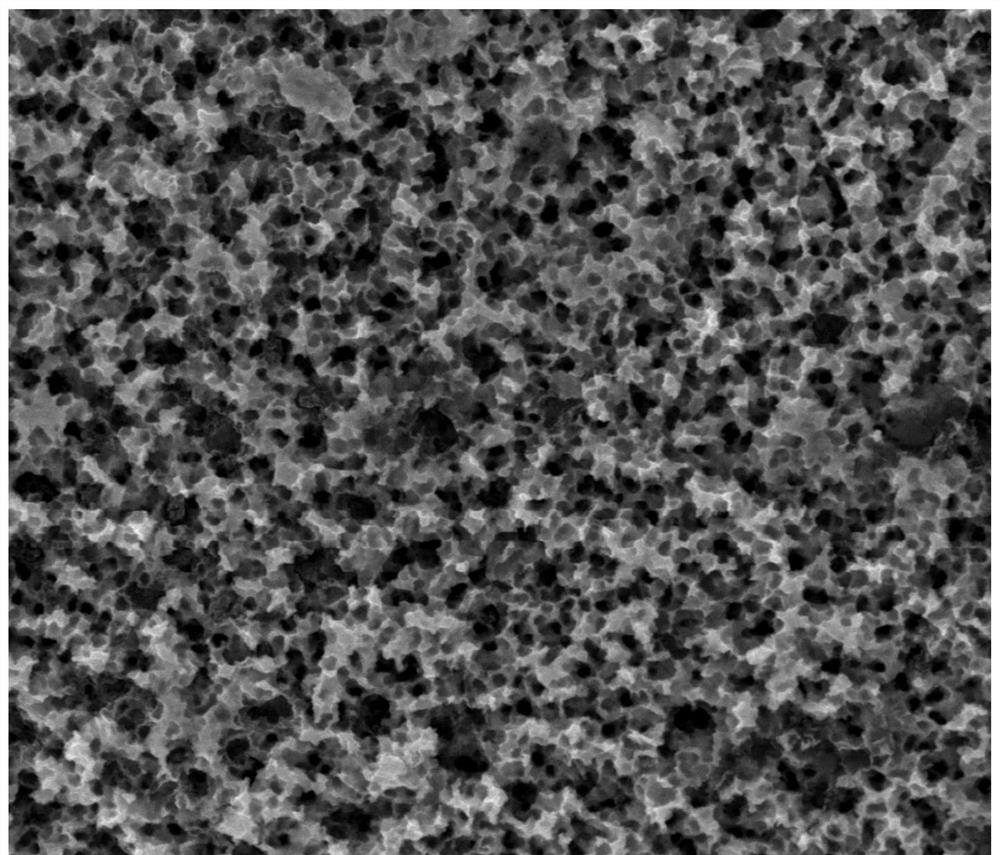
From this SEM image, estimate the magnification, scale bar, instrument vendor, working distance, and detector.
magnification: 20 K X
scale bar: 5000 nm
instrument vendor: FEI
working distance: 10.7 mm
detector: SE